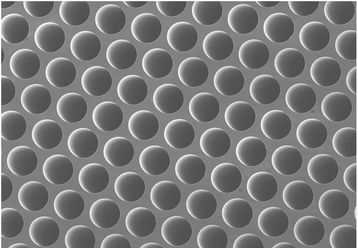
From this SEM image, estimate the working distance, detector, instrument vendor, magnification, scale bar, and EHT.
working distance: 10.4 mm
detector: SE(M)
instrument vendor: Hitachi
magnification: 0.5 K X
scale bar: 100000 nm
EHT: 10 kV